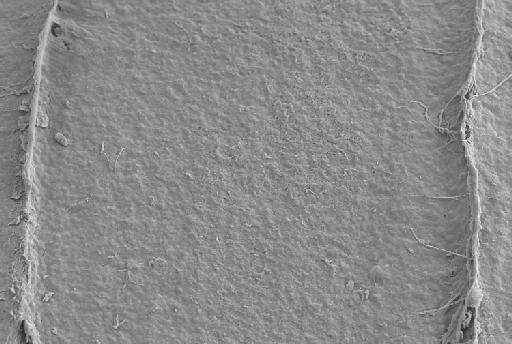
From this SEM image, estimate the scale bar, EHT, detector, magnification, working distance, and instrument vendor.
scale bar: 10000 nm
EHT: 5 kV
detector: SE2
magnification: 3.91 K X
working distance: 5.7 mm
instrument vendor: Zeiss